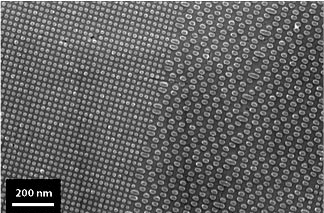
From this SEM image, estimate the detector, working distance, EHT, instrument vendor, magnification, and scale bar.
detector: InLens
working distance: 3 mm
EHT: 5 kV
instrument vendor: Zeiss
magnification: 60 K X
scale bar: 100 nm